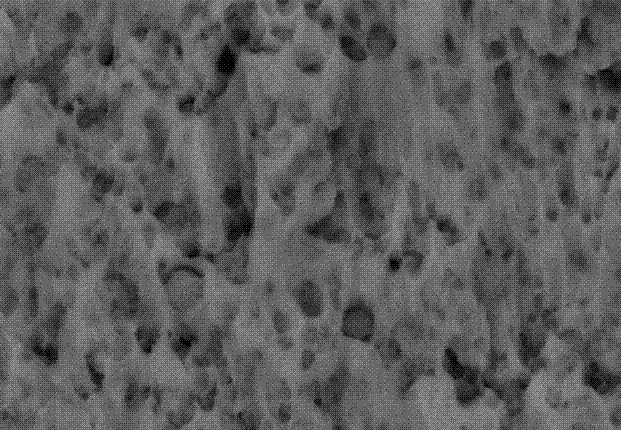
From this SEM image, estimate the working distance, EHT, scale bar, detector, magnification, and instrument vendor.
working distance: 8.5 mm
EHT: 15 kV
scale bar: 200 nm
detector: AsB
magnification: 50 K X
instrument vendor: Zeiss